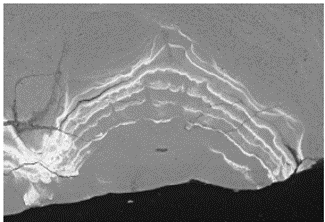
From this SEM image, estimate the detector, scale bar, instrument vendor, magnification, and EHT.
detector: BSE-COMP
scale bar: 50000 nm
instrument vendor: Hitachi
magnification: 0.7 K X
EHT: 20 kV